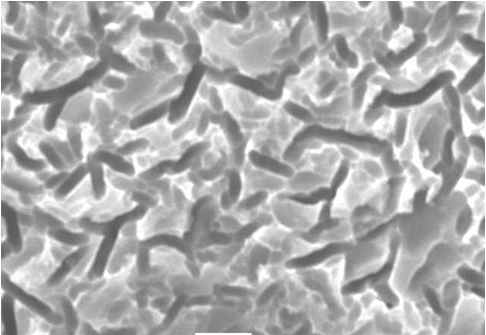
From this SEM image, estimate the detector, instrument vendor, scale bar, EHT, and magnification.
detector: SEI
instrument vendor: JEOL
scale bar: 5000 nm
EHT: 20 kV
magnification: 3 K X